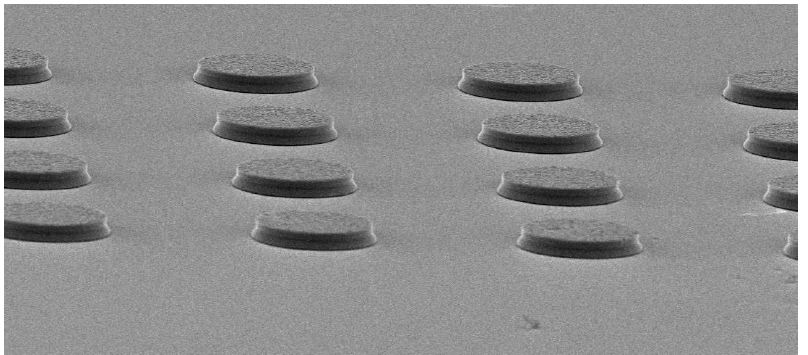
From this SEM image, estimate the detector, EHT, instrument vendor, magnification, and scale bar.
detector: SEI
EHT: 20 kV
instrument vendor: JEOL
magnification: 0.55 K X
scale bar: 20000 nm